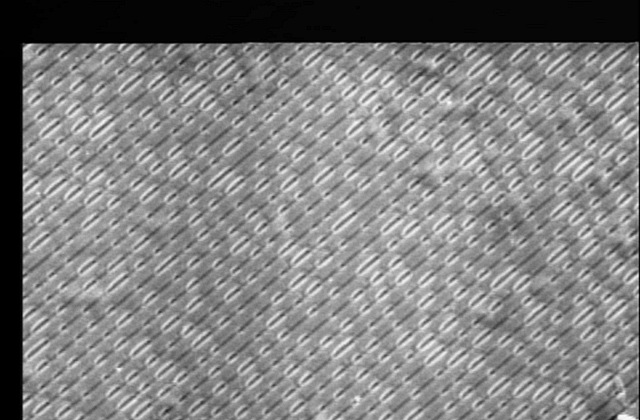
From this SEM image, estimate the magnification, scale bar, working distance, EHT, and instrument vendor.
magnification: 2.2 K X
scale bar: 10000 nm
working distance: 9 mm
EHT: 20 kV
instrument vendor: JEOL